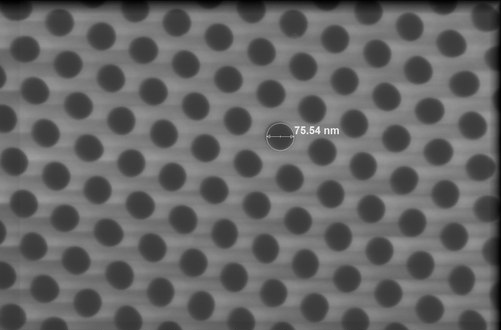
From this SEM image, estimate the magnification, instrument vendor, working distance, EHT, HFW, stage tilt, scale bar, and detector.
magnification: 150 K X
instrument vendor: FEI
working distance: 10 mm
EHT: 5 kV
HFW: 1.38 µm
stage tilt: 0°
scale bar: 300 nm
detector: ICE